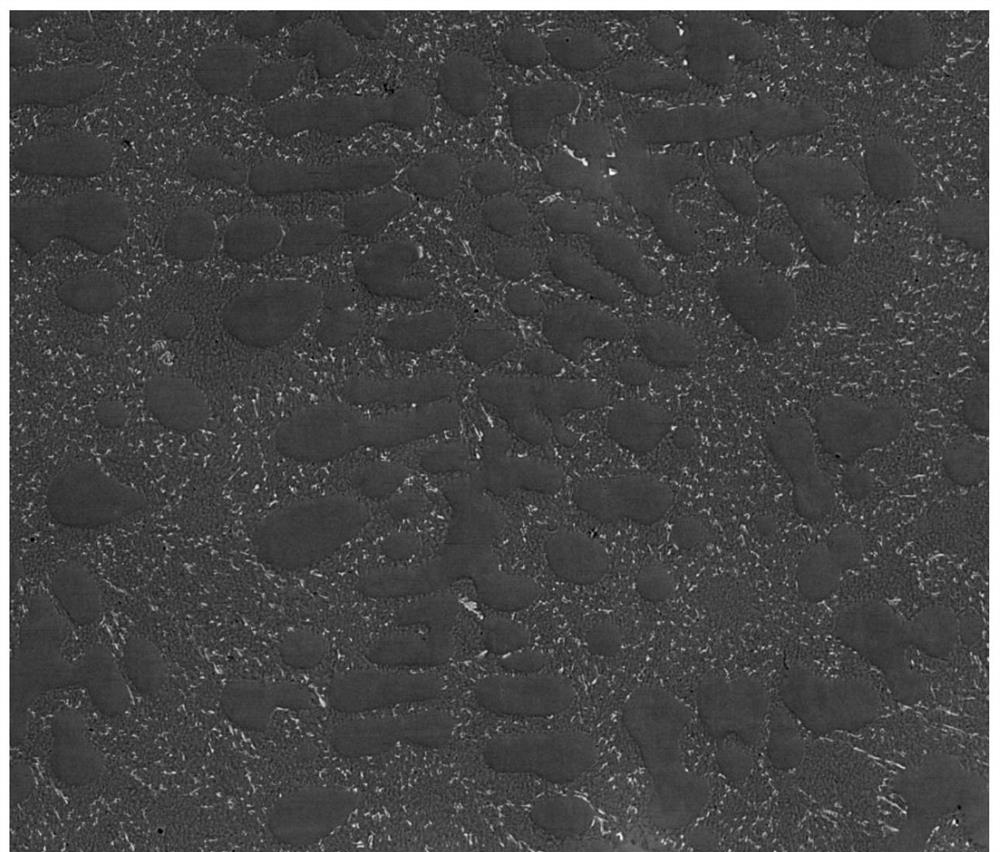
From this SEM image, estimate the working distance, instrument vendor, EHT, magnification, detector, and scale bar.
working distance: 12.8 mm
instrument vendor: FEI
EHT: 20 kV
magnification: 0.5 K X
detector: DualBSD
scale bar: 200000 nm